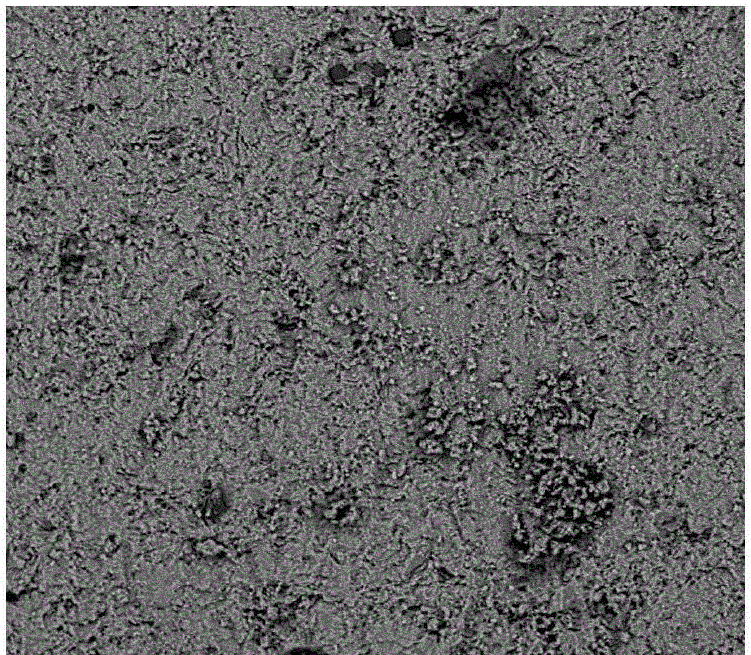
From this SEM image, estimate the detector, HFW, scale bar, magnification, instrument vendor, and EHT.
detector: BSD Full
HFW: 77.5 µm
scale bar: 20000 nm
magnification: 3.5 K X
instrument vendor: Thermo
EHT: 5 kV